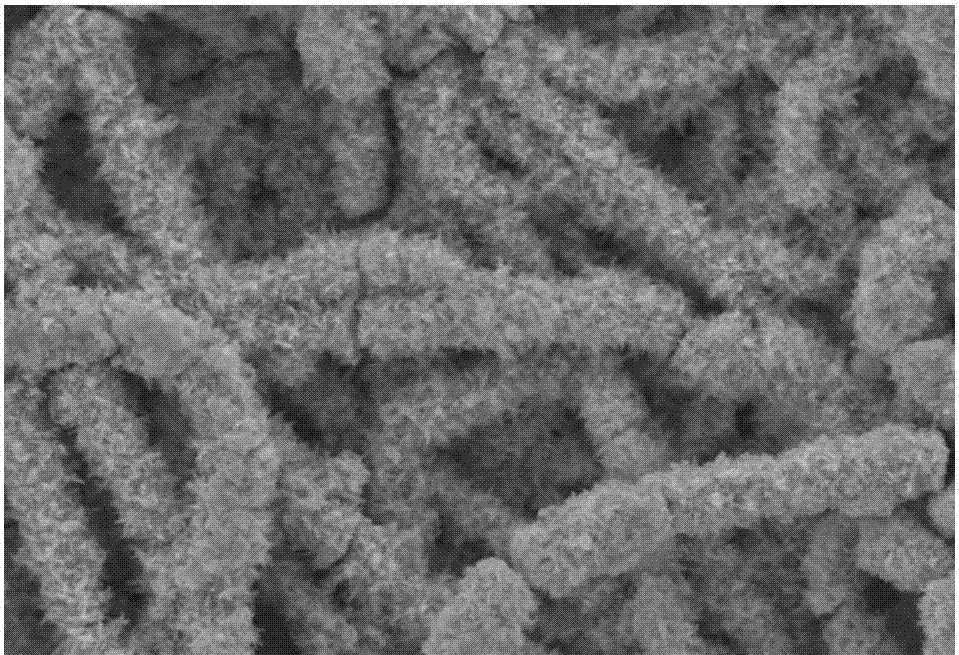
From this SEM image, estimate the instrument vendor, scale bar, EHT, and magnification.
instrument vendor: JEOL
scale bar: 10000 nm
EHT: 20 kV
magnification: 2 K X